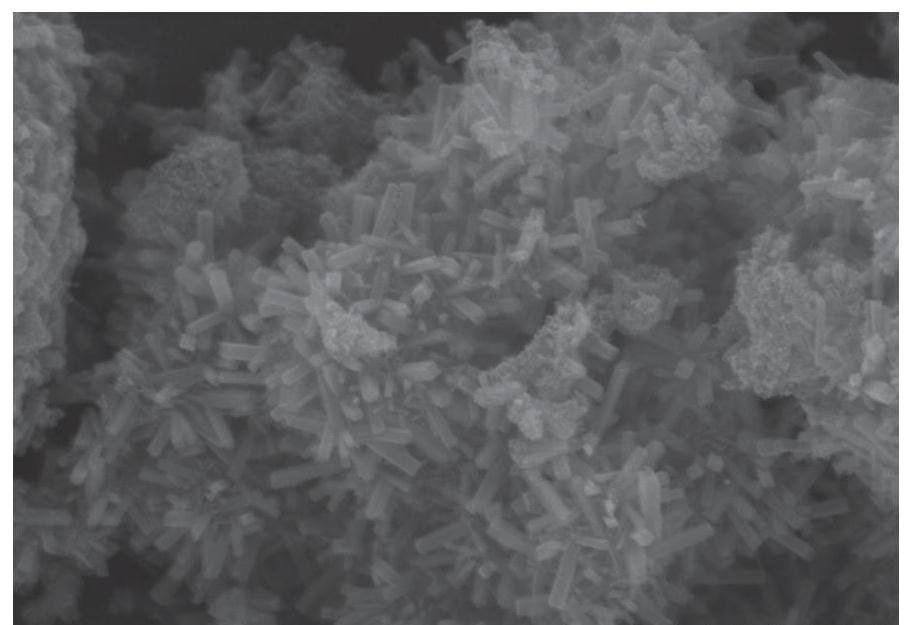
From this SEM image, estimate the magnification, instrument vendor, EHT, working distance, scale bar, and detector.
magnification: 50 K X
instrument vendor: Hitachi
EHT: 10 kV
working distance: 8.9 mm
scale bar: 1000 nm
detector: SE(M)